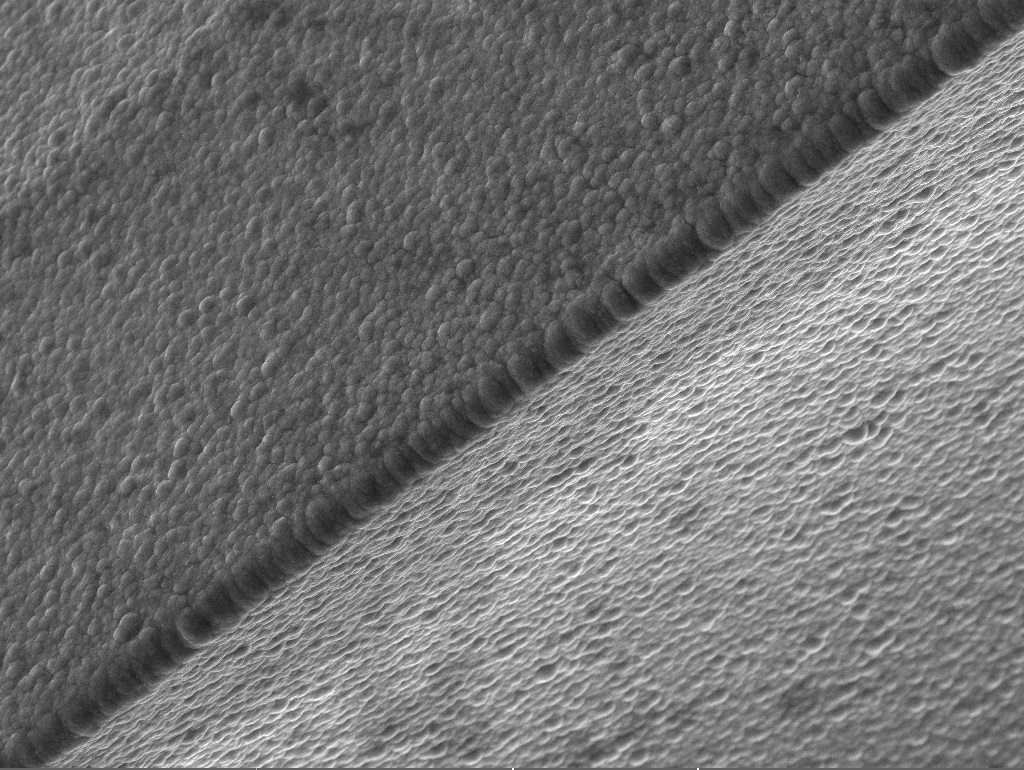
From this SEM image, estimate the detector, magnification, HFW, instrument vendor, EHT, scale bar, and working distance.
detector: SE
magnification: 1 K X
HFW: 277 µm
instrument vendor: Tescan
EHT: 20 kV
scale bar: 50000 nm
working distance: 9.91 mm